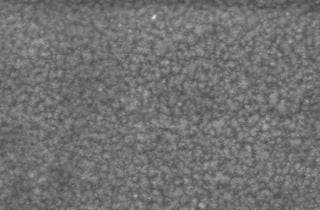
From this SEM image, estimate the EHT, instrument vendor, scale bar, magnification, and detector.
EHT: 15 kV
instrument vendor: JEOL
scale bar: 5000 nm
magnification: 5 K X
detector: SEI